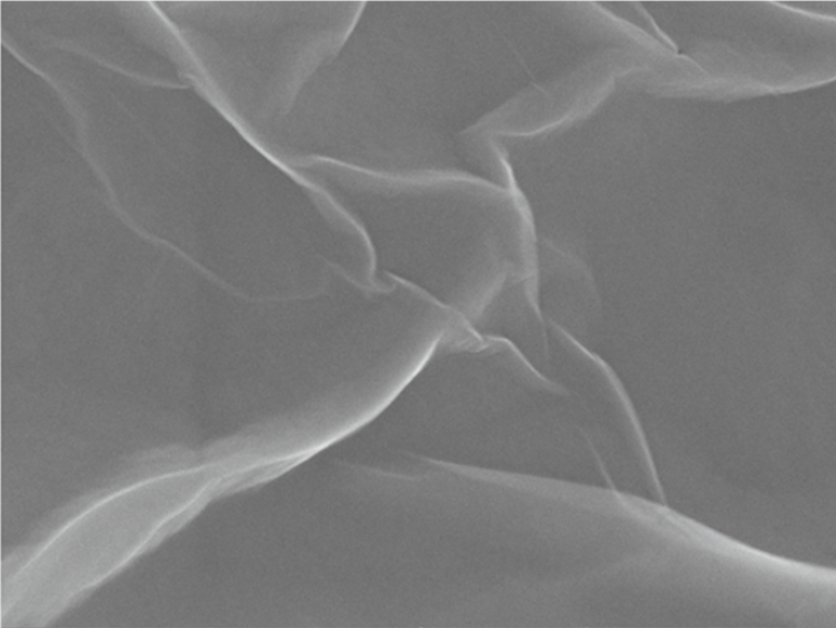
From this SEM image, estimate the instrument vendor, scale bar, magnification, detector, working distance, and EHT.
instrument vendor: Tescan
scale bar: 1000 nm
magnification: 50 K X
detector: In-Beam SE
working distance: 6 mm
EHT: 20 kV